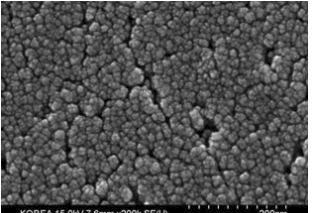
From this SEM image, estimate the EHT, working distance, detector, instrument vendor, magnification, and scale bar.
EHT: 15 kV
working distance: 7.6 mm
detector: SE(U)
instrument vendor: Hitachi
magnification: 200 K X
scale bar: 200 nm